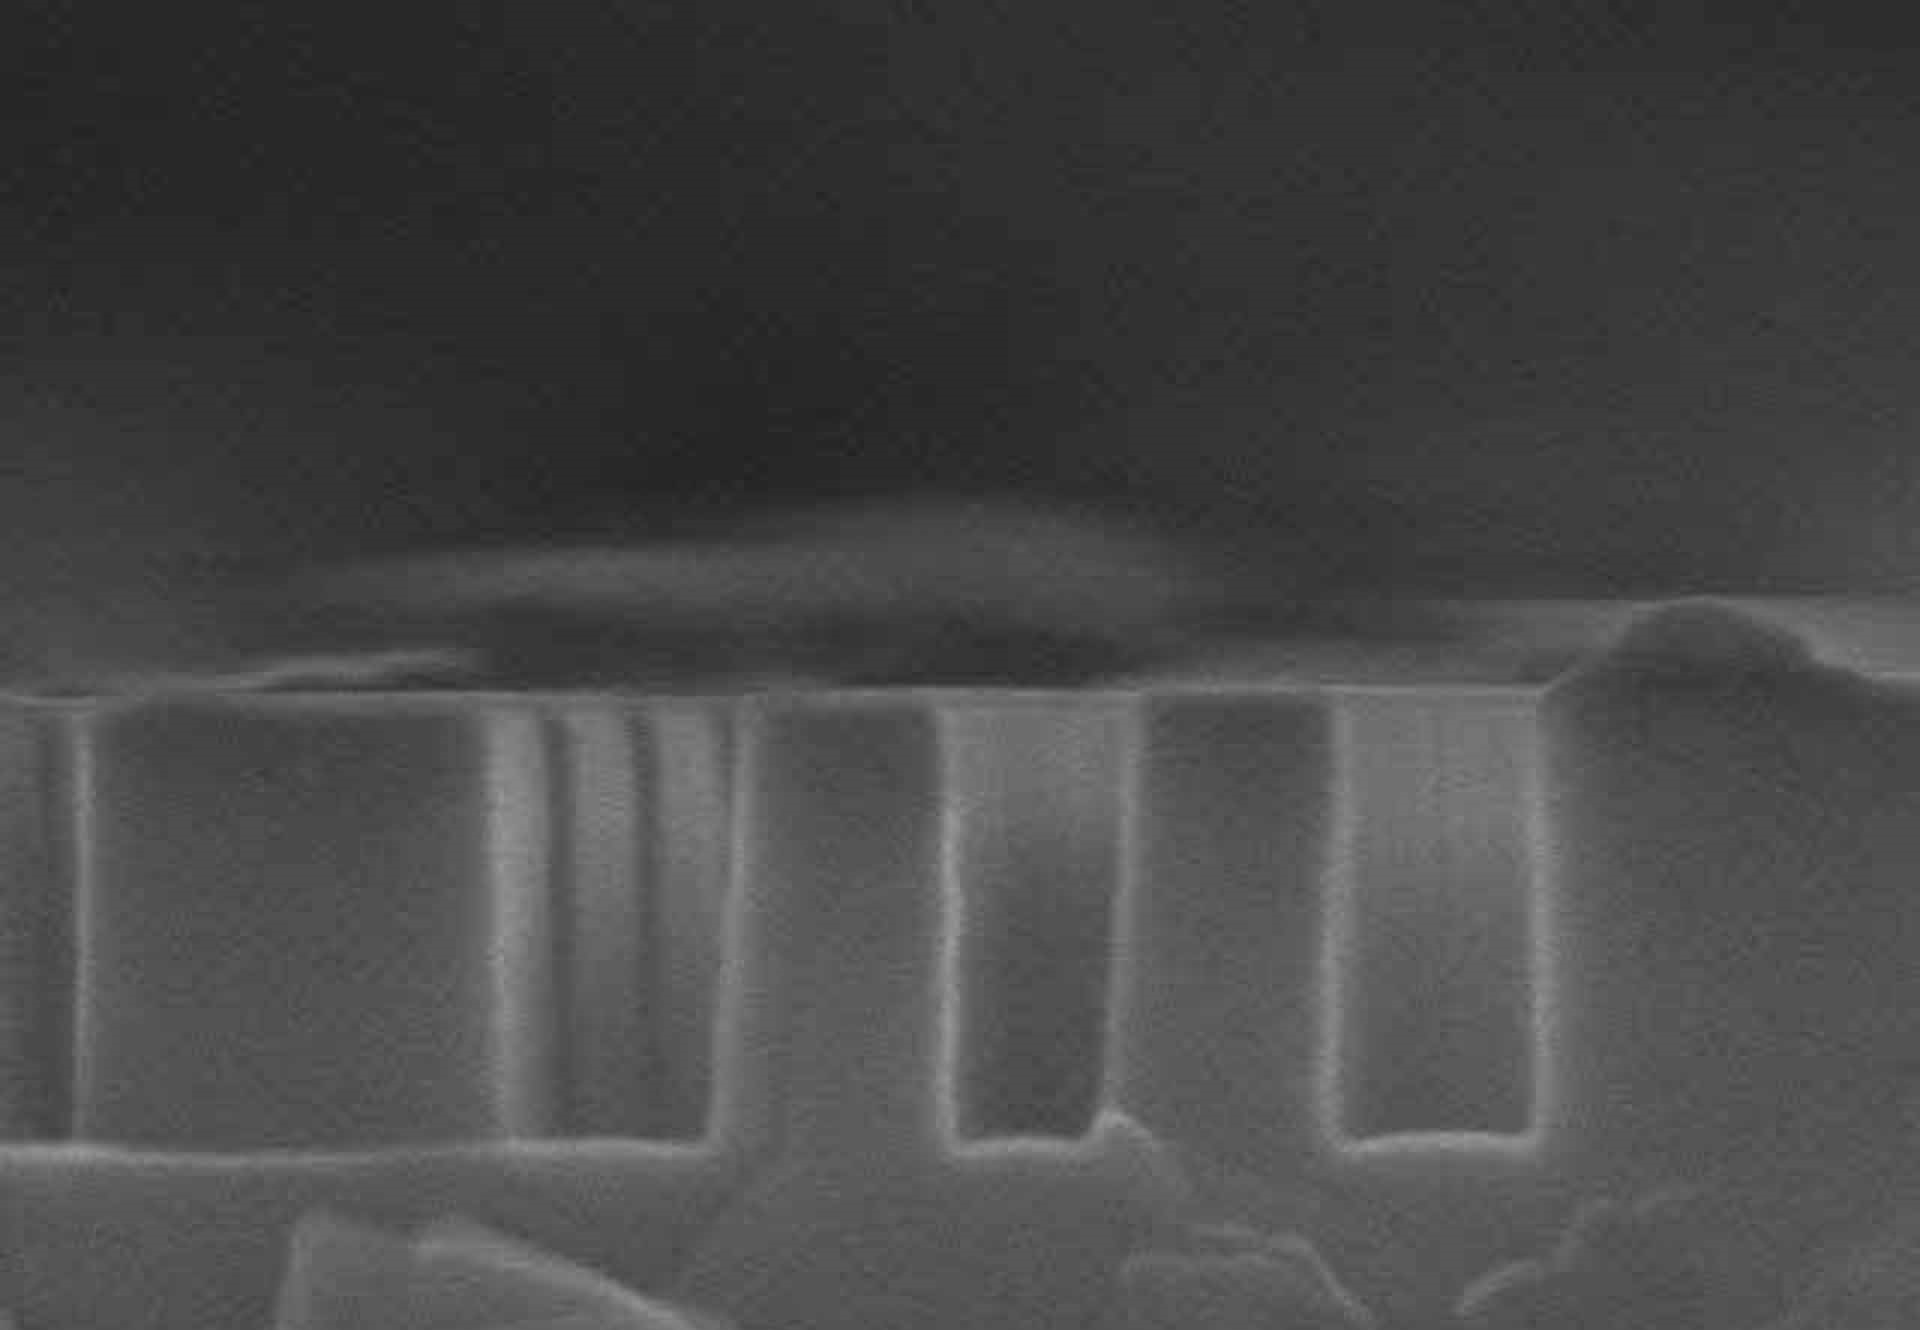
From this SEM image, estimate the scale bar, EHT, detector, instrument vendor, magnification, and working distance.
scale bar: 500 nm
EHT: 15 kV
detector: SE(M)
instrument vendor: Hitachi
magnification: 100 K X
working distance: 15.3 mm